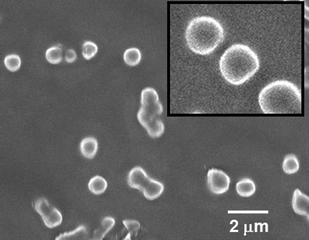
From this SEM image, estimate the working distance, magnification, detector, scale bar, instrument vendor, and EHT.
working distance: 7.9 mm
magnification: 7 K X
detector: SEI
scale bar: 1000 nm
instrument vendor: JEOL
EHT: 5 kV